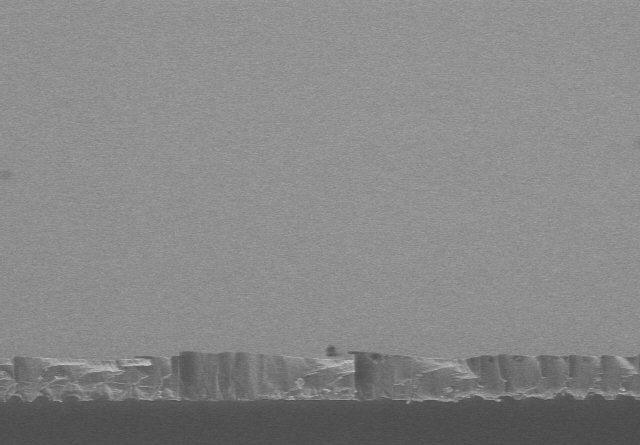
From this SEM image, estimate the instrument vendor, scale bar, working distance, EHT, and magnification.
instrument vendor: Hitachi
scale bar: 20000 nm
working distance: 10.2 mm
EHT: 5 kV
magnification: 2 K X